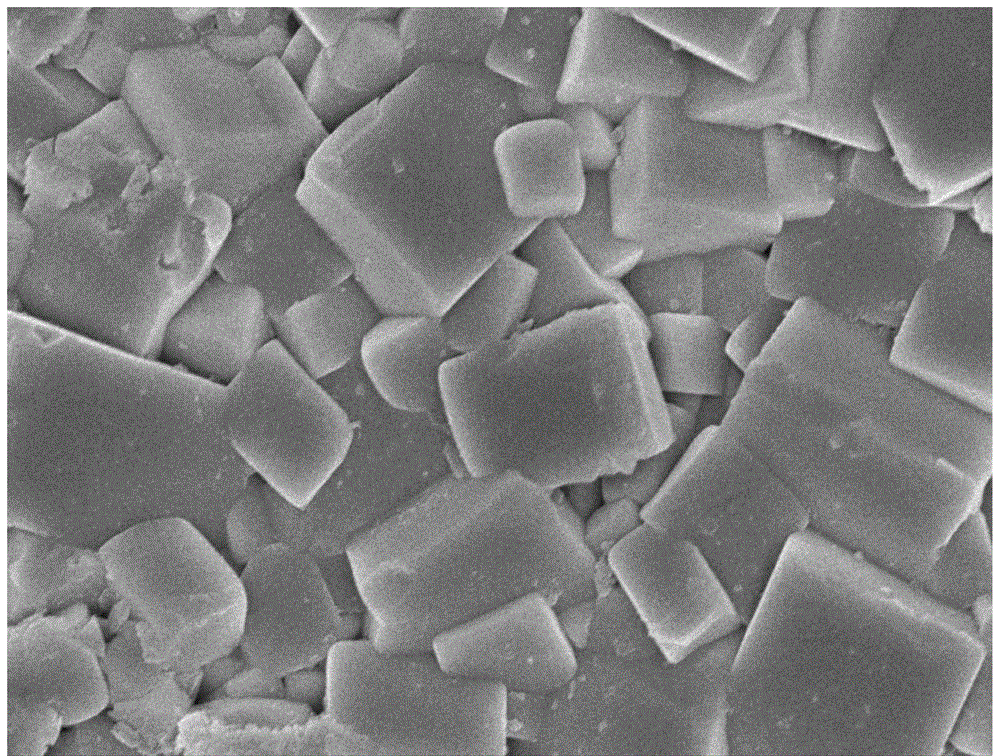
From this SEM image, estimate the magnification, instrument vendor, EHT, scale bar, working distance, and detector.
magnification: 15 K X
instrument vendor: JEOL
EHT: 10 kV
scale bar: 1000 nm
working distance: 8.7 mm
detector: SEI